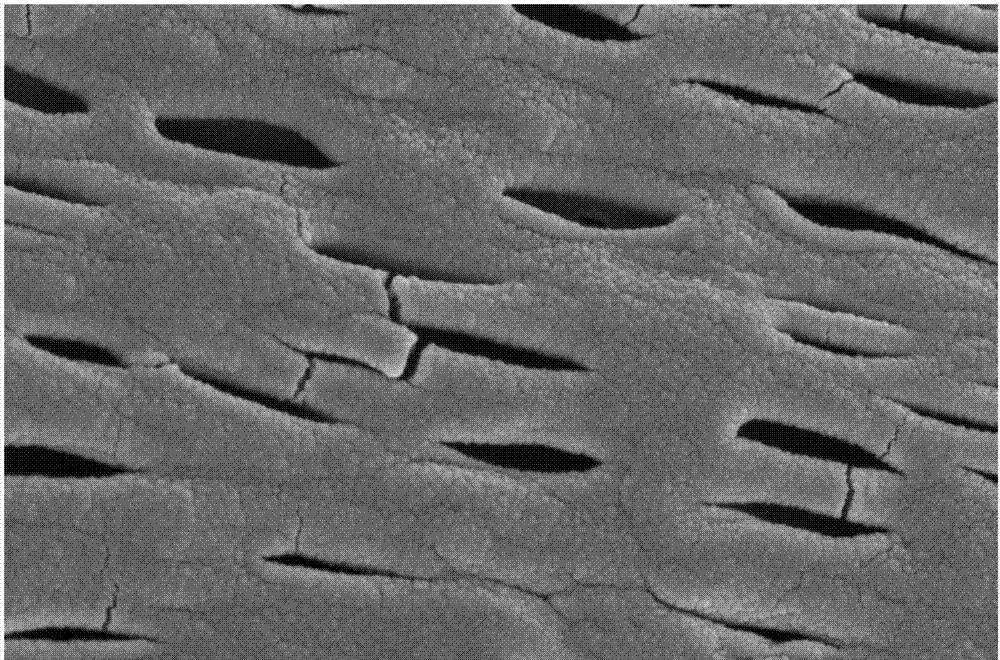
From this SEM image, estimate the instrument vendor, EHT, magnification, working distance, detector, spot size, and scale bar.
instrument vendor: FEI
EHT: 5 kV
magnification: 30 K X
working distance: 4.6 mm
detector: TLD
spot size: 3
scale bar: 1000 nm